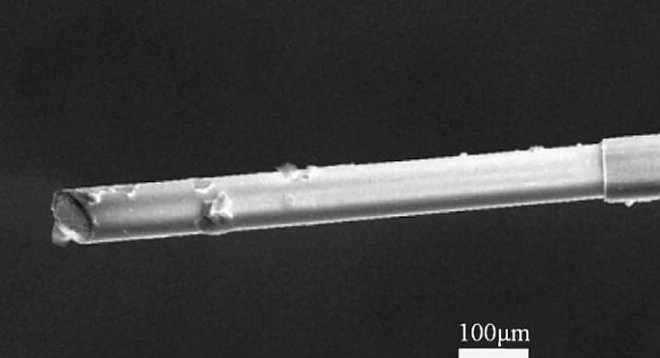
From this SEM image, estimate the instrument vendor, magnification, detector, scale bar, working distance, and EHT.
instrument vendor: FEI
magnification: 0.5 K X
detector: SE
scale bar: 100000 nm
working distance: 14.2 mm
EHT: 20 kV